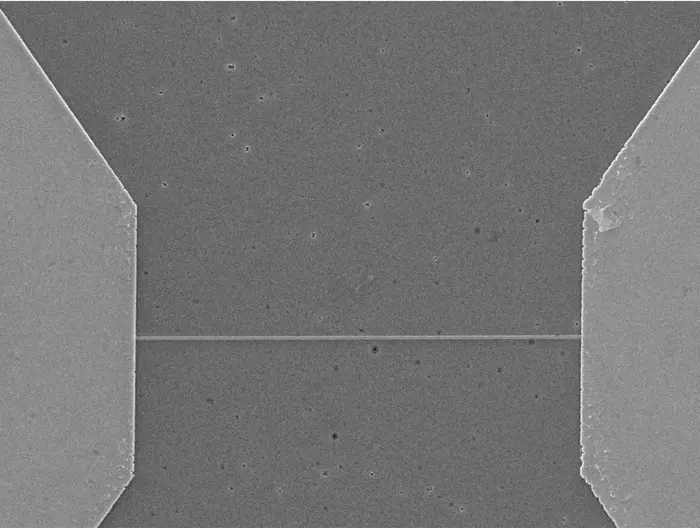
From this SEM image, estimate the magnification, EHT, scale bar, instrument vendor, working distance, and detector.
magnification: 1.5 K X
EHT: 15 kV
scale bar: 10000 nm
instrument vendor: JEOL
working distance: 6.1 mm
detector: SEI